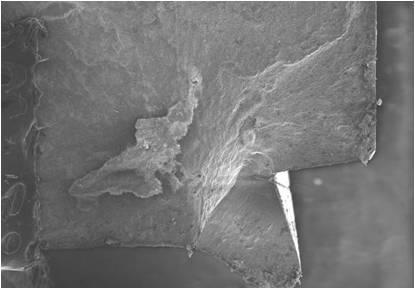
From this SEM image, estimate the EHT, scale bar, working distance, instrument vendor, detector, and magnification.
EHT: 15 kV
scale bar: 1000000 nm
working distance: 15 mm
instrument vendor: Hitachi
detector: SE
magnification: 0.035 K X